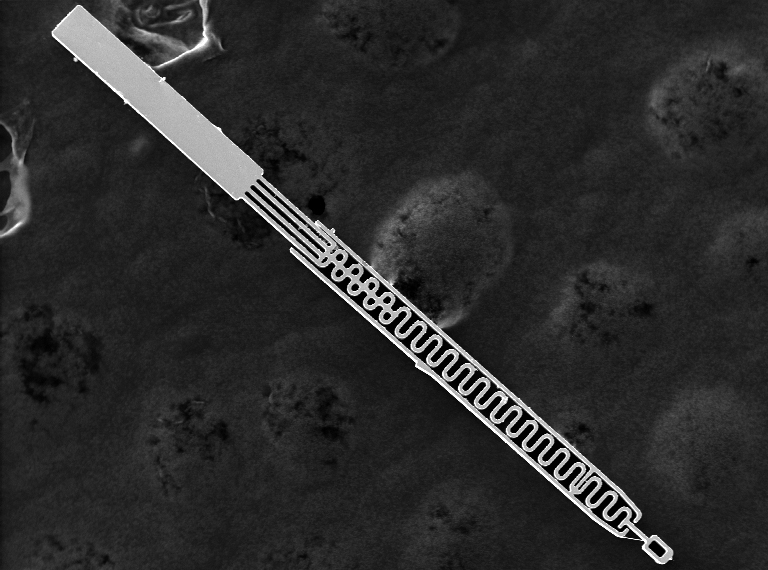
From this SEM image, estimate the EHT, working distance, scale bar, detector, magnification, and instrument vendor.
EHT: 20 kV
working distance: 10 mm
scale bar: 300000 nm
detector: SEI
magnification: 0.2 K X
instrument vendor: JEOL